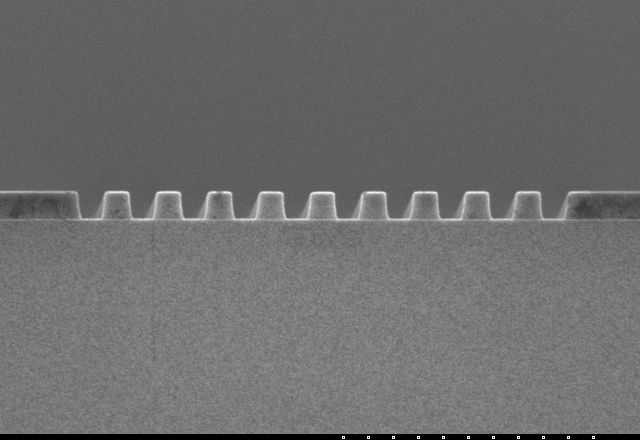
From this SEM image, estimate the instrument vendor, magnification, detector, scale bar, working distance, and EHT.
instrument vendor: Hitachi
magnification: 5 K X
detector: SE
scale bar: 10000 nm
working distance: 8 mm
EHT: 5 kV